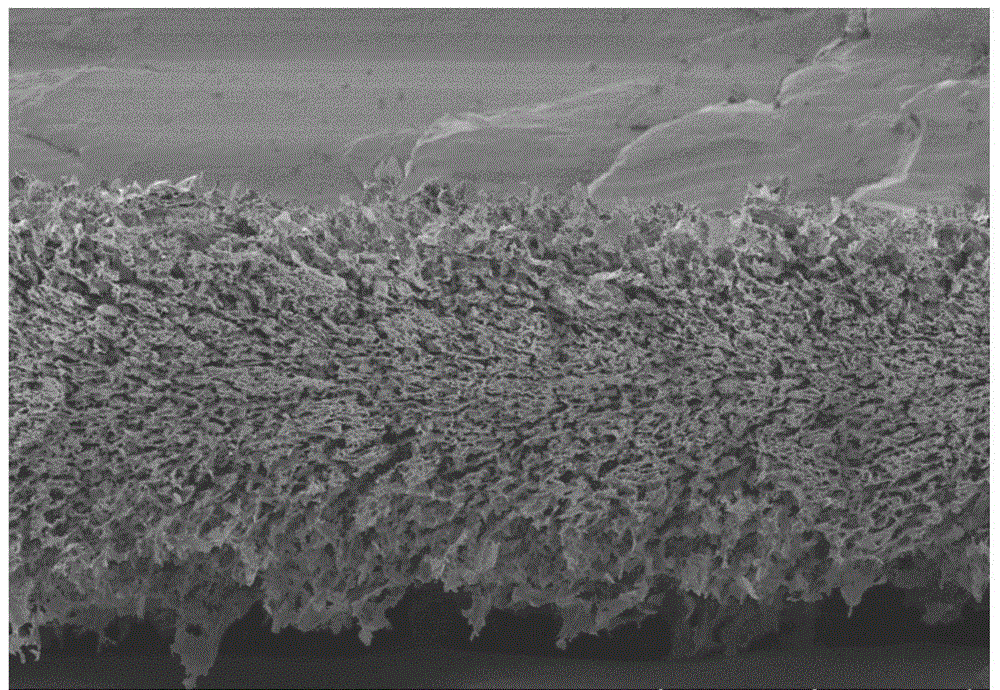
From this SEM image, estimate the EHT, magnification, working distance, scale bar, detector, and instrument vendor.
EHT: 3 kV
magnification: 0.2 K X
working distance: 9.9 mm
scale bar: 200000 nm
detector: LM(UL)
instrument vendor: Hitachi